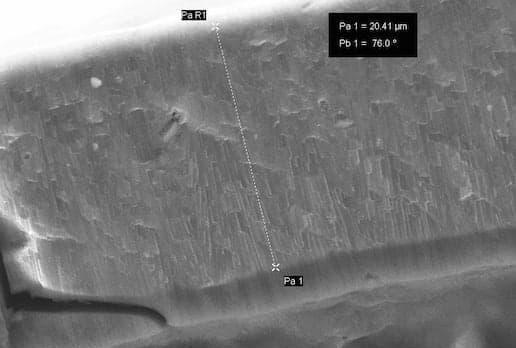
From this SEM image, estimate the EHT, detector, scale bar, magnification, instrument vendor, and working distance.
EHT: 20 kV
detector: InLens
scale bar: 2000 nm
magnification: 6.5 K X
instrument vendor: Zeiss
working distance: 7.7 mm